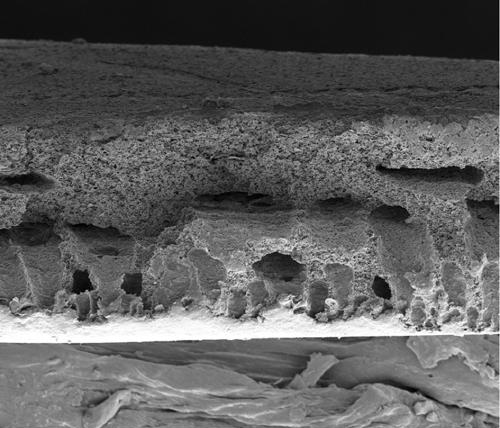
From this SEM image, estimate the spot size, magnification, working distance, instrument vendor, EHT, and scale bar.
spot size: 3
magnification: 1.2 K X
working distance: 4.7 mm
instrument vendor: FEI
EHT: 3 kV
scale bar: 50000 nm